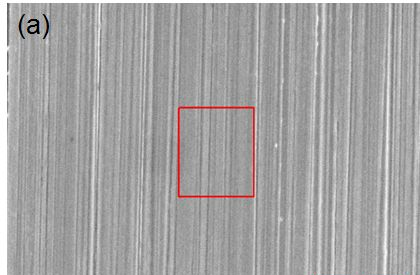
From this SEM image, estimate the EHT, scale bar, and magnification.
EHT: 15 kV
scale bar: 10000 nm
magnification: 3 K X